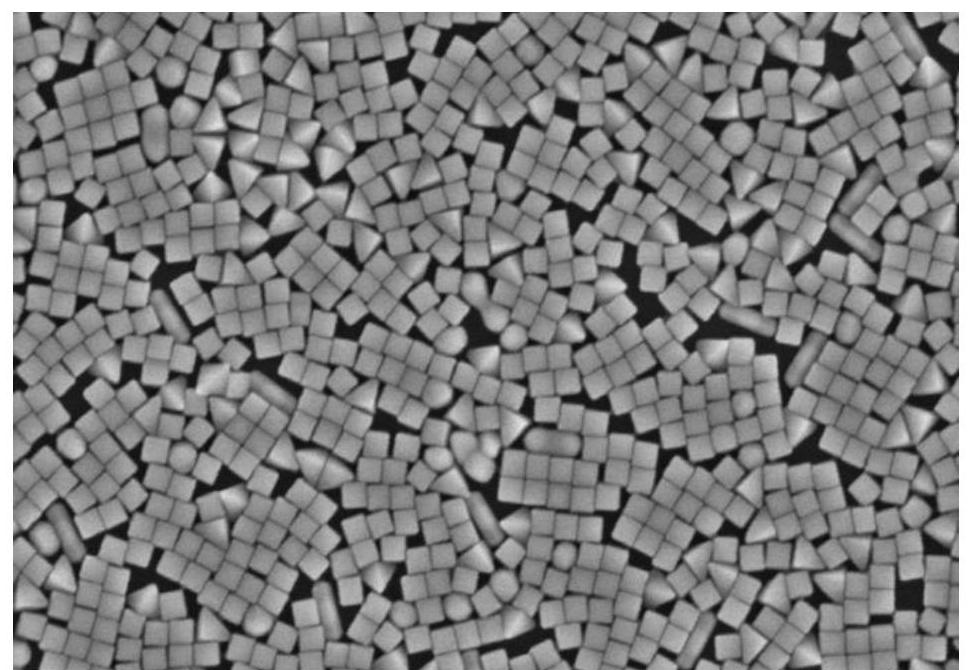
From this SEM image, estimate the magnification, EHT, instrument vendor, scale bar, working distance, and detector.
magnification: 60 K X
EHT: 5 kV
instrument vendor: Hitachi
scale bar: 500 nm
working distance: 12 mm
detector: SE(M)